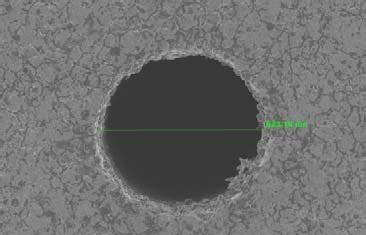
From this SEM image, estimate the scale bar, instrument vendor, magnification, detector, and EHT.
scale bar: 200000 nm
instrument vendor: JEOL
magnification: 0.095 K X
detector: SEI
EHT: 10 kV